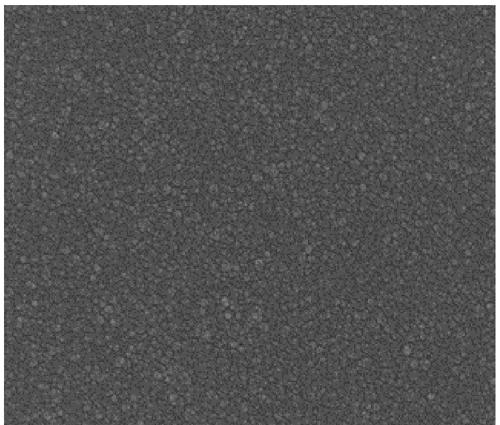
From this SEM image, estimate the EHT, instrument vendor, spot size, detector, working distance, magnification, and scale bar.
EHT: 10 kV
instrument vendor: FEI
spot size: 3.5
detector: TLD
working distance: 5 mm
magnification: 100 K X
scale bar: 1000 nm